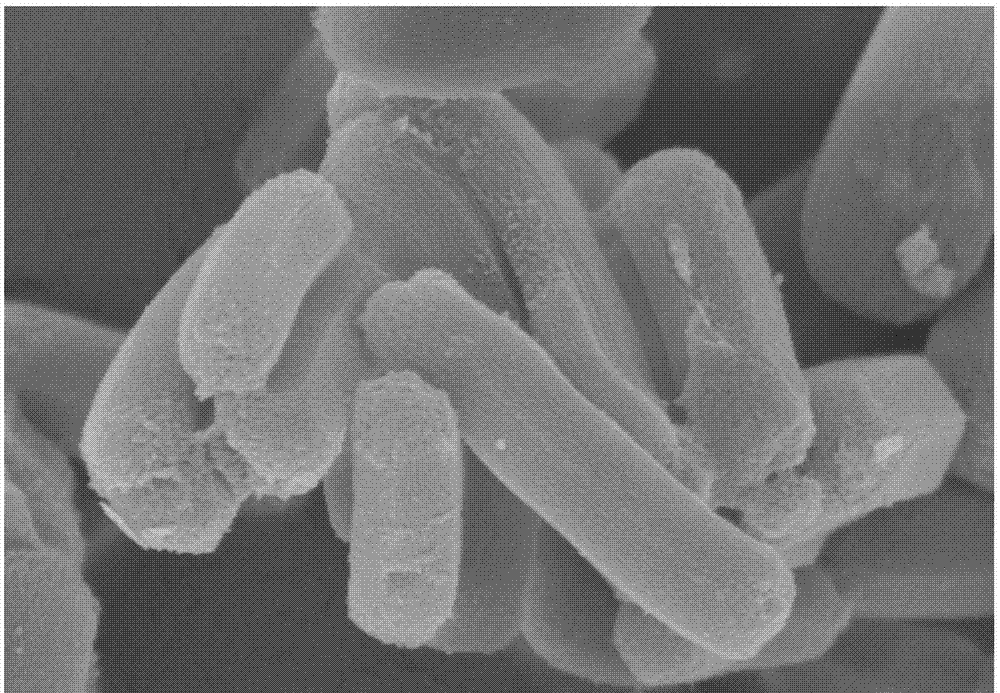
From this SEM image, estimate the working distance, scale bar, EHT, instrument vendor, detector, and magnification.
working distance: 8.3 mm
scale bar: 1000 nm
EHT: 3 kV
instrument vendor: Hitachi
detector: SE(M)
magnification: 45 K X